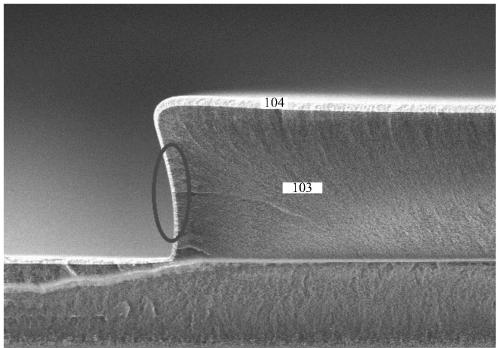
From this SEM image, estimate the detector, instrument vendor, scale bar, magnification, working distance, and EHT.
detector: SE(M)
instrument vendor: Hitachi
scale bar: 4000 nm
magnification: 12 K X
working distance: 10.8 mm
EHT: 3 kV